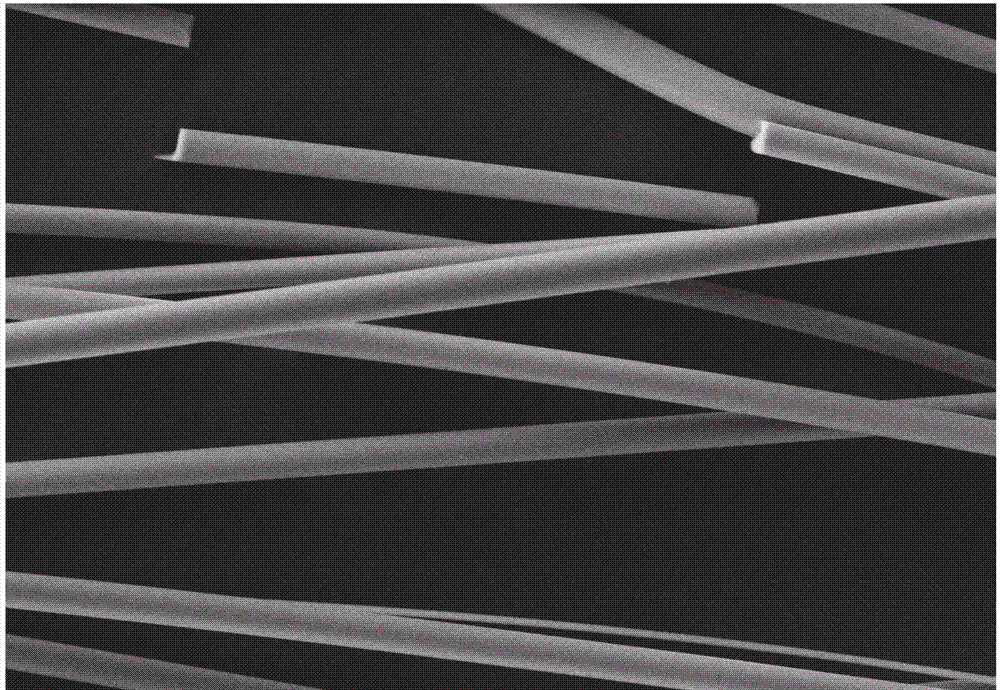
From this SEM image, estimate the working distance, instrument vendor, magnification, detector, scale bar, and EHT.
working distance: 15 mm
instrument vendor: Hitachi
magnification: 0.5 K X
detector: SE(M)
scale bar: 100000 nm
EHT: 20 kV